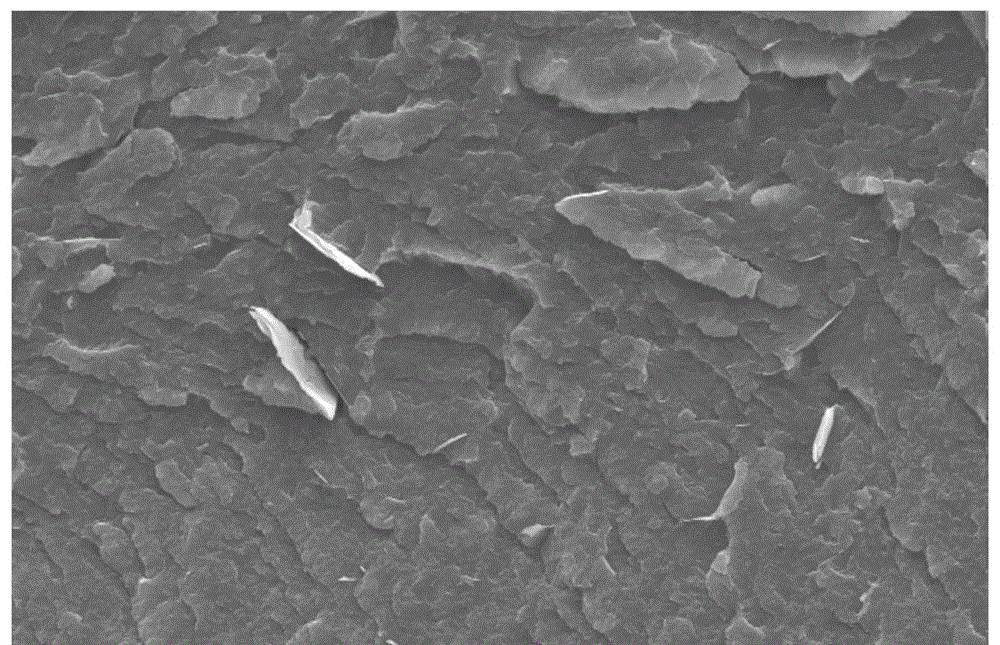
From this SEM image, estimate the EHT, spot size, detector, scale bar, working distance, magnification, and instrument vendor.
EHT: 10 kV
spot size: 4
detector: SE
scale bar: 10000 nm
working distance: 6.4 mm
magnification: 5 K X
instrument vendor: FEI